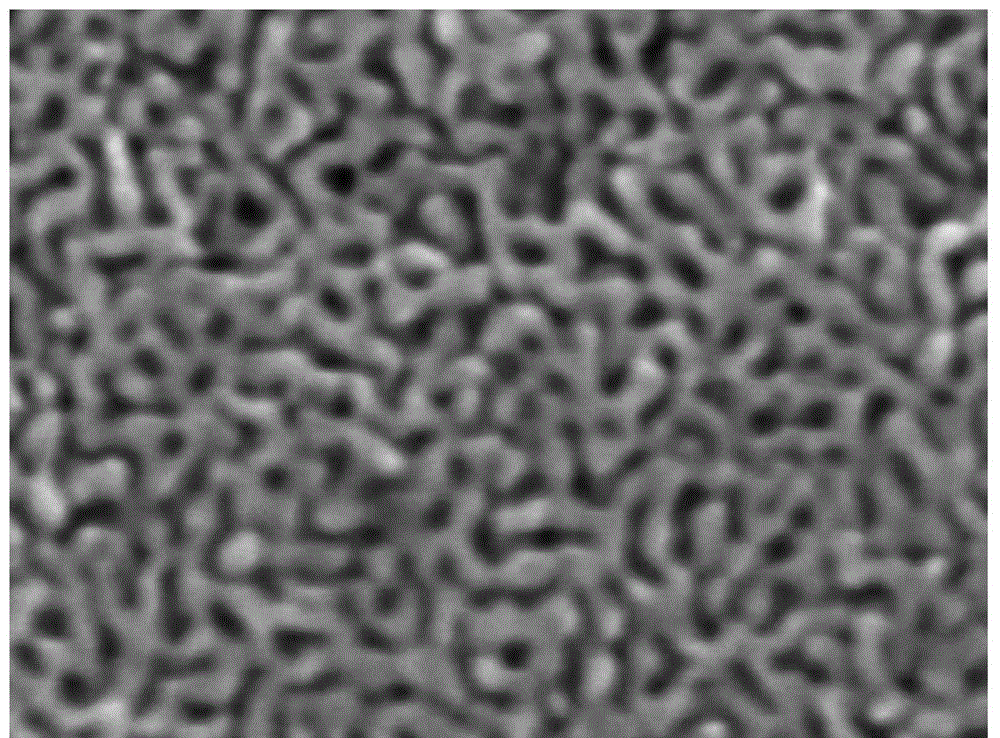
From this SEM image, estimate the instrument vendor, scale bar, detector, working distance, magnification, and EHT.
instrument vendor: JEOL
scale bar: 10 nm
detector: UED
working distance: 4 mm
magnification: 300 K X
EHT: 10 kV